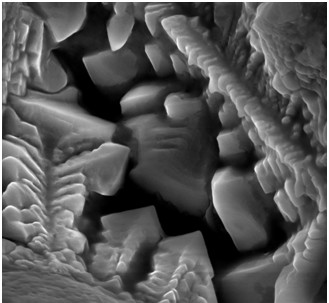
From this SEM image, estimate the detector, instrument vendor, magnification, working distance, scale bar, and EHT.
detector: SE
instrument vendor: other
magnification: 20 K X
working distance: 9.2 mm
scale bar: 2000 nm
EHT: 10 kV